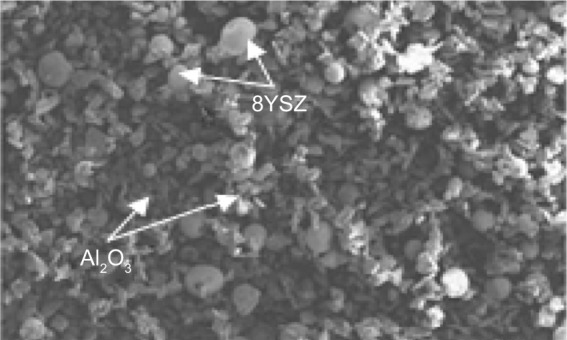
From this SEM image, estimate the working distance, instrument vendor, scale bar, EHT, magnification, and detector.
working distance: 14.3 mm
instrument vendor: JEOL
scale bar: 500000 nm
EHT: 15 kV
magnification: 0.1 K X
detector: SE